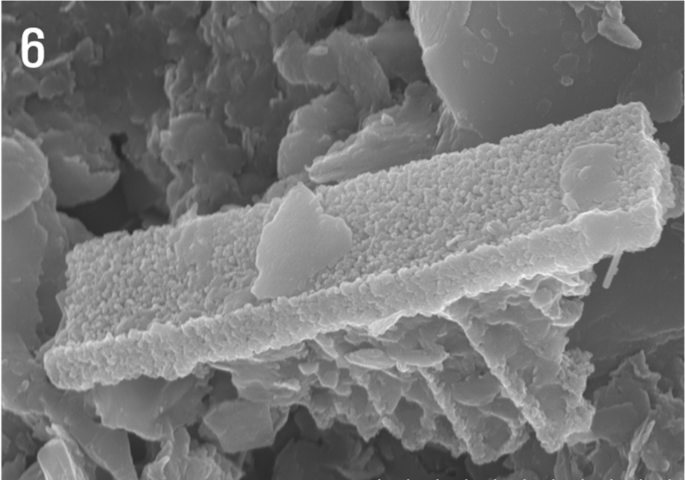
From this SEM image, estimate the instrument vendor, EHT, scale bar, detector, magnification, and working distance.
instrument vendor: Hitachi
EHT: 15 kV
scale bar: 3000 nm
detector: SE(U)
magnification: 18 K X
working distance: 11.8 mm